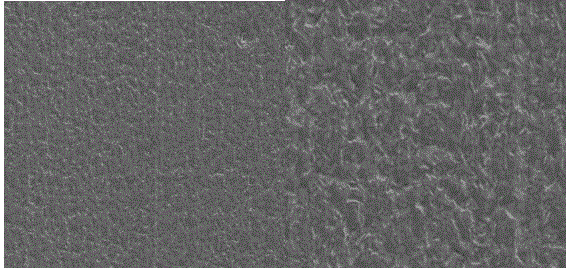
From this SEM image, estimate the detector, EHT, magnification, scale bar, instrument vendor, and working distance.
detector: SE2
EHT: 2 kV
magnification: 20 K X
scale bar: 2000 nm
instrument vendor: Zeiss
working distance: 11.3 mm